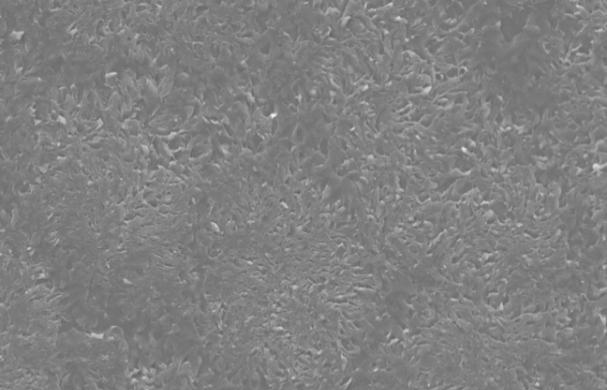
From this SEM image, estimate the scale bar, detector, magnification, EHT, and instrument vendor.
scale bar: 50000 nm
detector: SEI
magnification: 0.5 K X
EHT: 15 kV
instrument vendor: JEOL